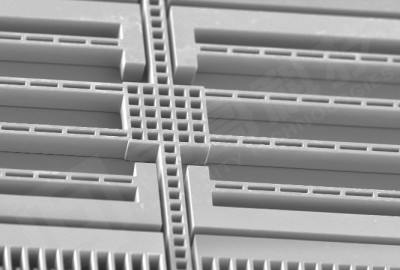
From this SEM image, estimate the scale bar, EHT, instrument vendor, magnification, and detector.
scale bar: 200000 nm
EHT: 10 kV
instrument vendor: JEOL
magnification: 0.09 K X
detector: SEI